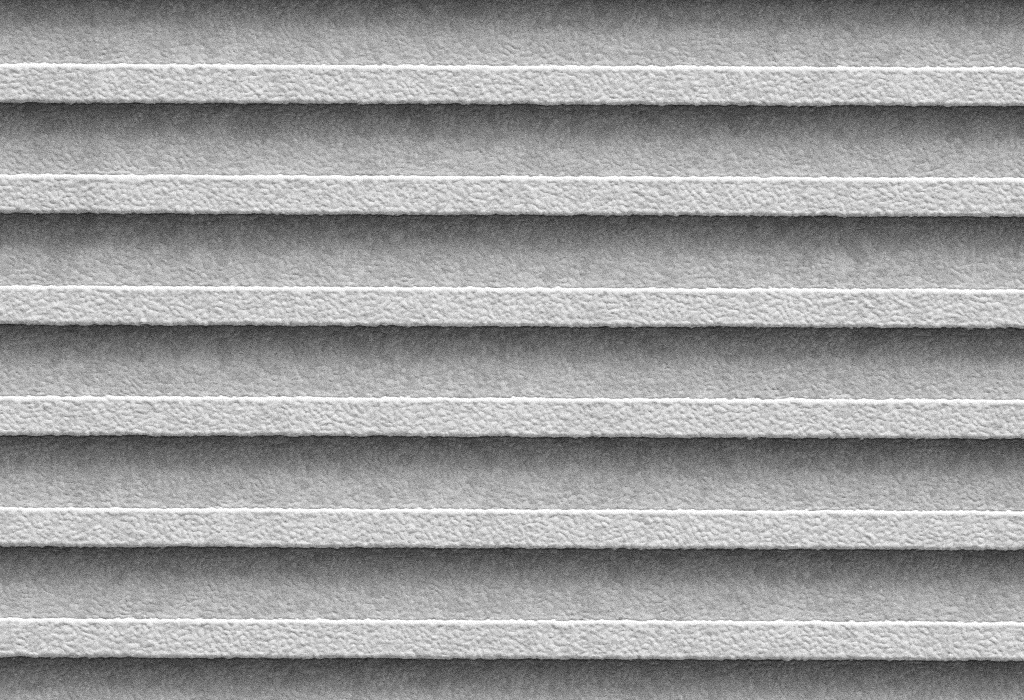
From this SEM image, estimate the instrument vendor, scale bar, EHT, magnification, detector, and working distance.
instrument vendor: Zeiss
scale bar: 1000 nm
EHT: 1 kV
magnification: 30 K X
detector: SE2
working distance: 6 mm